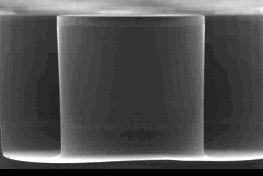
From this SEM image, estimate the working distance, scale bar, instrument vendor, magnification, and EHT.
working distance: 24 mm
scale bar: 100000 nm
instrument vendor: JEOL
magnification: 0.16 K X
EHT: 15 kV